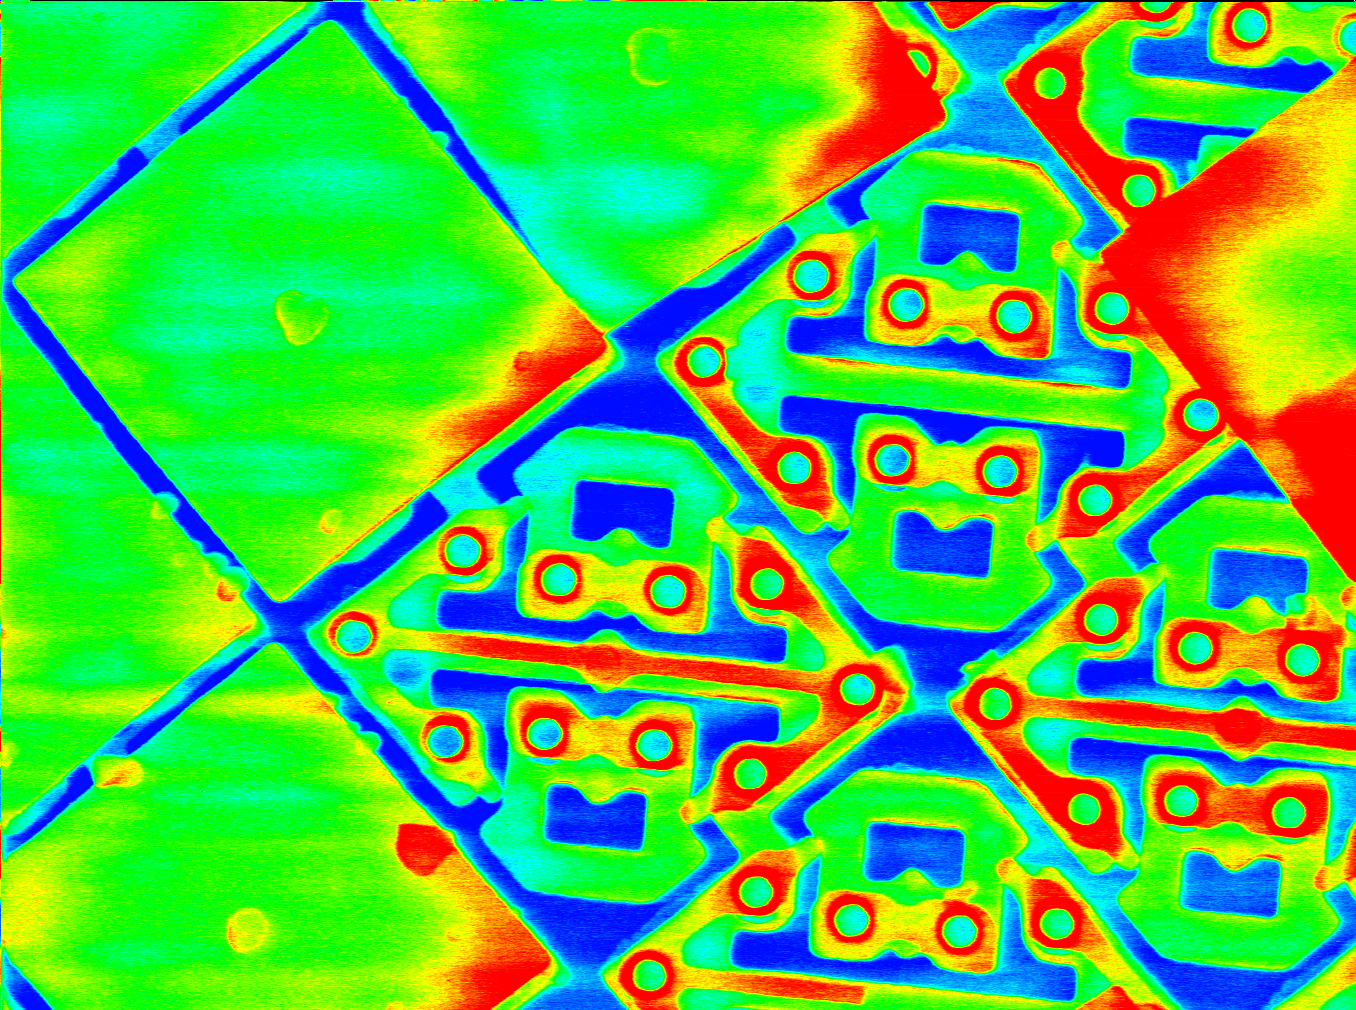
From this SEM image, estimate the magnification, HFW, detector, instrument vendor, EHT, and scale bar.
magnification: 3 K X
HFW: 40 µm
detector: SE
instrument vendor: other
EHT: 15 kV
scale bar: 3330 nm